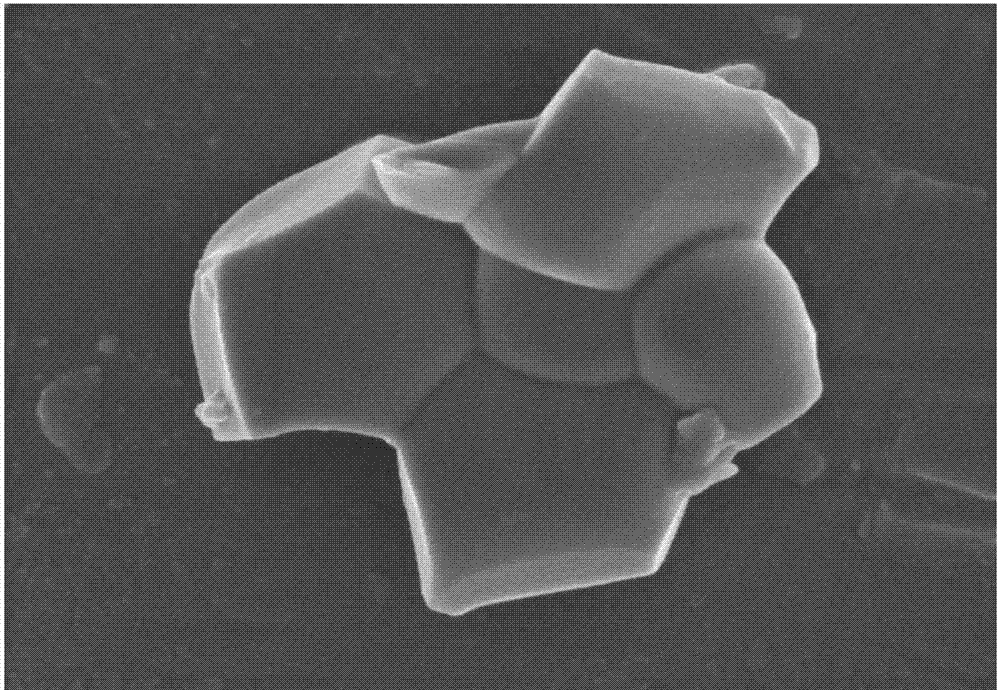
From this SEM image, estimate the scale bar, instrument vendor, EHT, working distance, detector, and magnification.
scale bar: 1000 nm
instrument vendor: Hitachi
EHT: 3 kV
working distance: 9.7 mm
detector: SE(M)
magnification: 40 K X